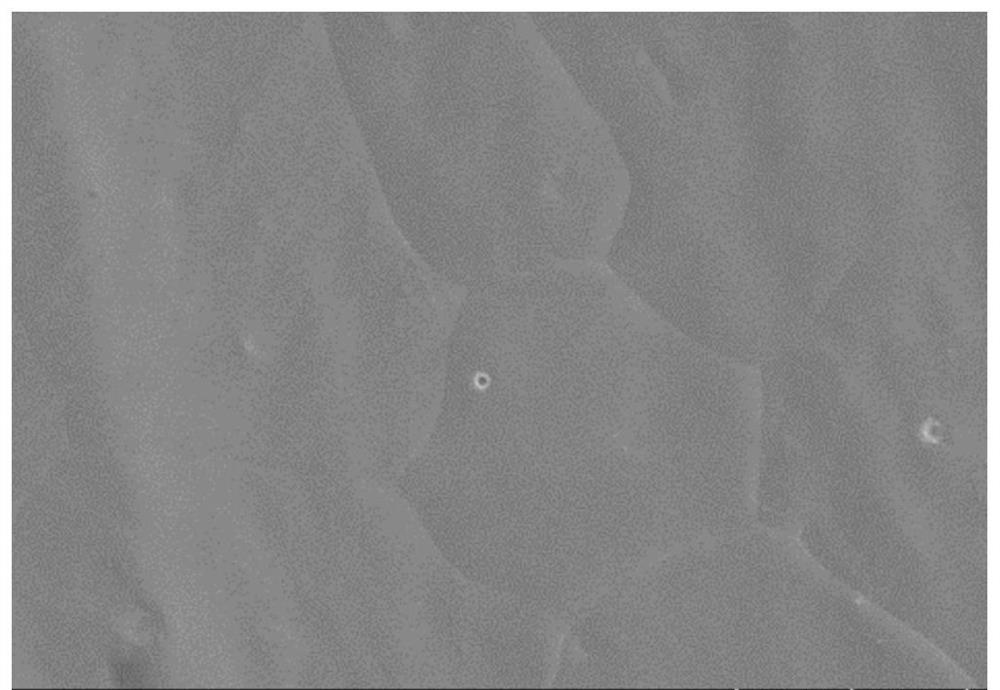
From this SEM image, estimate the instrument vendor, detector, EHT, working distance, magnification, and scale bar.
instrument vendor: Hitachi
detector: SE(UL)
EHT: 3 kV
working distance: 10.6 mm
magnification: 3 K X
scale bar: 10000 nm